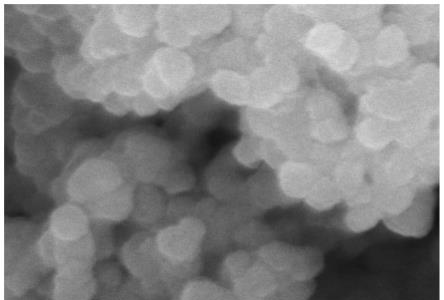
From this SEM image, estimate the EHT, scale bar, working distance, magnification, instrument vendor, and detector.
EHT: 15 kV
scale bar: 500 nm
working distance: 12.6 mm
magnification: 100 K X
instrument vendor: Hitachi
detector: SE(UL)